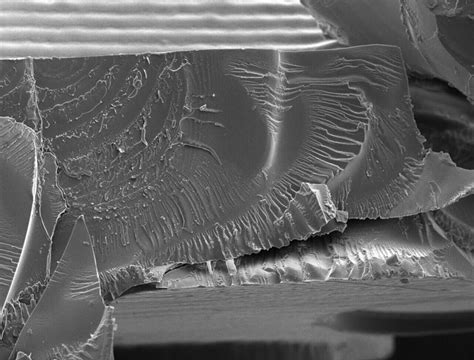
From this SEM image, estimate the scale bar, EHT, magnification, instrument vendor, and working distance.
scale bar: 1000000 nm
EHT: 20 kV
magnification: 0.04 K X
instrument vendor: JEOL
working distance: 15.2 mm